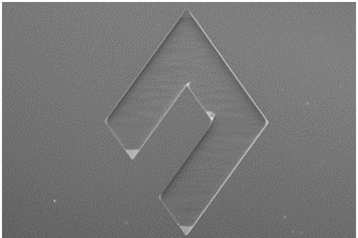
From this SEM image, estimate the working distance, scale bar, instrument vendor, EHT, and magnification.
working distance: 22.6 mm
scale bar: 500000 nm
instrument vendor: Hitachi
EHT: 15 kV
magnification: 0.08 K X